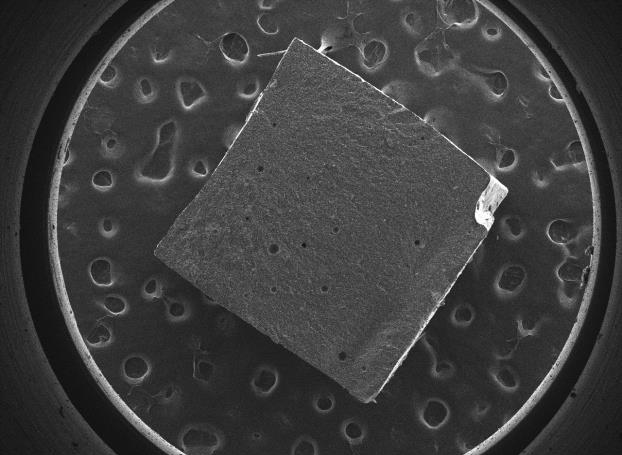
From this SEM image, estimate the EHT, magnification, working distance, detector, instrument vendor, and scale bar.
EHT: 10 kV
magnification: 0.025 K X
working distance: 14 mm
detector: SEI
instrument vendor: JEOL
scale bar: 1000000 nm